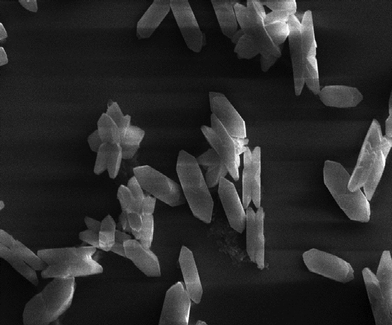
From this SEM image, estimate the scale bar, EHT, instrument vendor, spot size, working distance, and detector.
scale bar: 2000 nm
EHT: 15 kV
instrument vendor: Zeiss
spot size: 350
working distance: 10.5 mm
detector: SE1